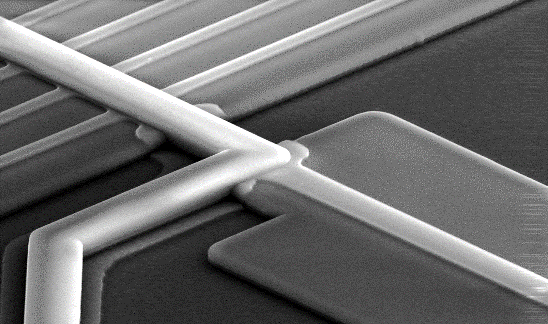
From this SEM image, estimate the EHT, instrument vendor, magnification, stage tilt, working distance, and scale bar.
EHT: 30 kV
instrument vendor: FEI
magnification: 0.8 K X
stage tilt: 52°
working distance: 10.2 mm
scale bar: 50000 nm